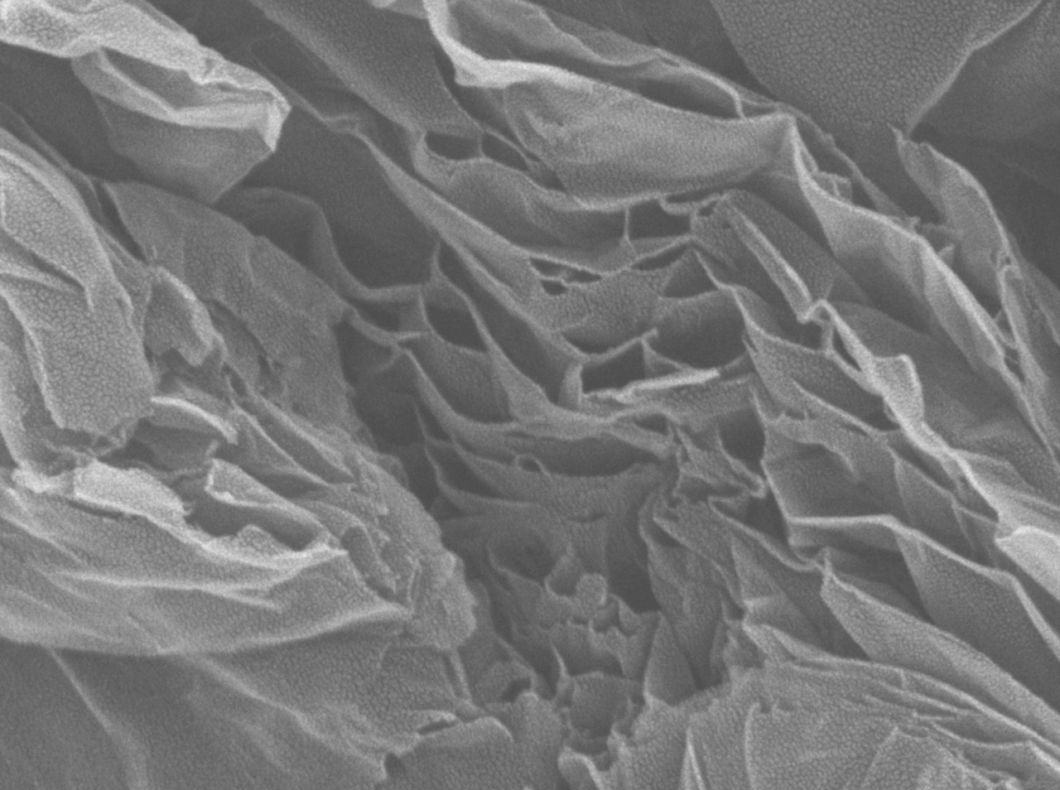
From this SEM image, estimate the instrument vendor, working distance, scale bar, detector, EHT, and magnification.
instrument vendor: JEOL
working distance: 6 mm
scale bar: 100 nm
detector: SEI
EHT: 5 kV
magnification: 50 K X